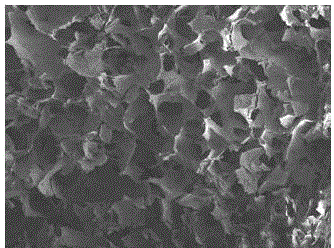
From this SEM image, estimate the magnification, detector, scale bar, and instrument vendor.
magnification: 0.2 K X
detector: ETD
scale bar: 500000 nm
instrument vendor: FEI